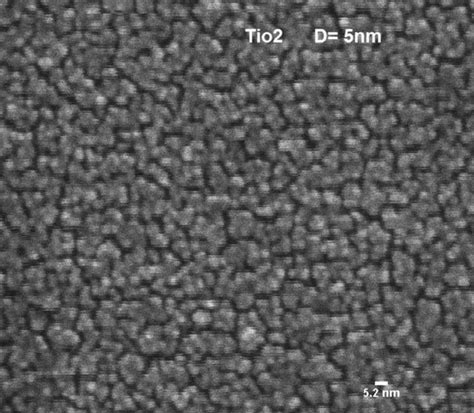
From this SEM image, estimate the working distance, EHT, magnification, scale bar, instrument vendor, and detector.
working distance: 5.18 mm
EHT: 15 kV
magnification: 350 K X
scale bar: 100 nm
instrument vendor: Tescan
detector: SE